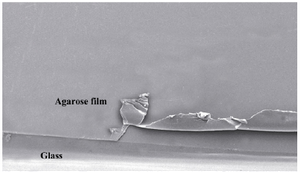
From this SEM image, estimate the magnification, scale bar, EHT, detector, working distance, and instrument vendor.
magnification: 0.034 K X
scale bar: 1000000 nm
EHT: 10 kV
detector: SEI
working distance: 16.2 mm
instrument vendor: other